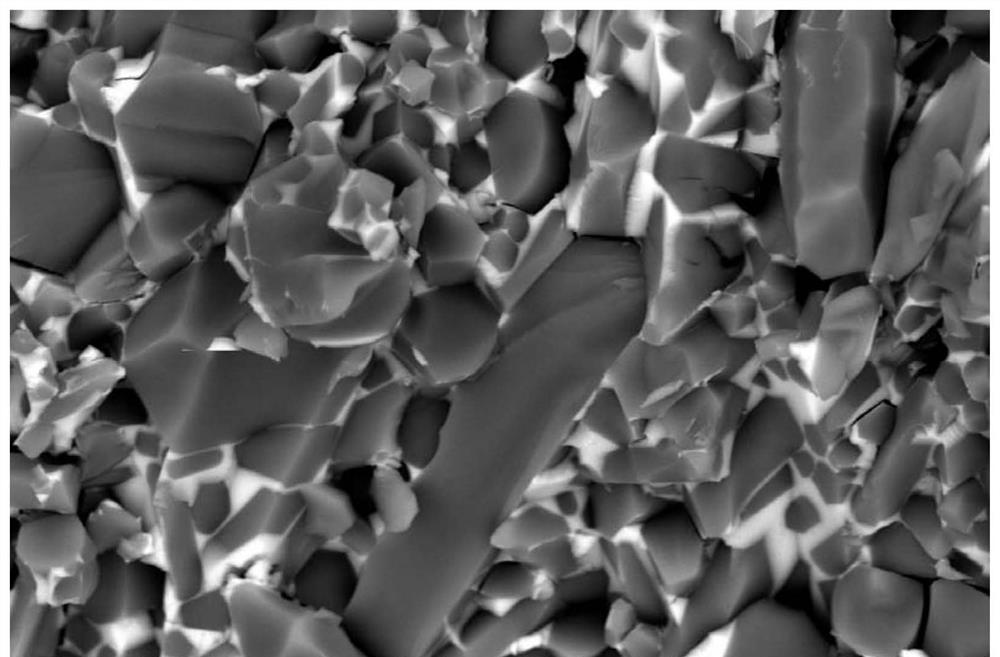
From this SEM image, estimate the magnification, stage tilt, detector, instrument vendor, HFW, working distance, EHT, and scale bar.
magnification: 8 K X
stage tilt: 0°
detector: CBS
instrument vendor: FEI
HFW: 15.9 µm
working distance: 4.3 mm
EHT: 10 kV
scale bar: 4000 nm